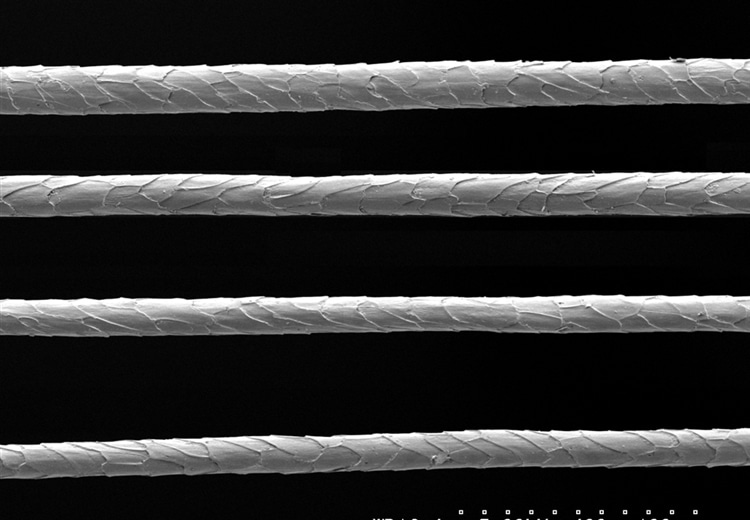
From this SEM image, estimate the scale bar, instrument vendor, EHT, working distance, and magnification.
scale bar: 100000 nm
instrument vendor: JEOL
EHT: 5 kV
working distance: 16.4 mm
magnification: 0.4 K X